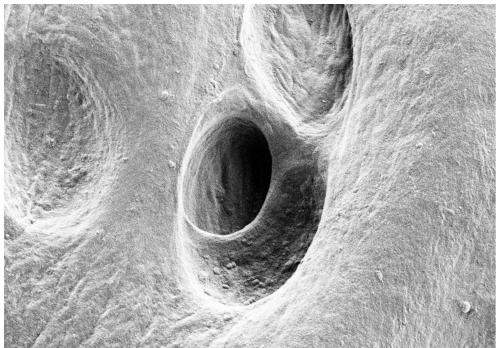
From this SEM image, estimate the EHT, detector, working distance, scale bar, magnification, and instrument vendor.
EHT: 1 kV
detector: SE2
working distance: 4.8 mm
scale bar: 10000 nm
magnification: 5 K X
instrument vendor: Zeiss